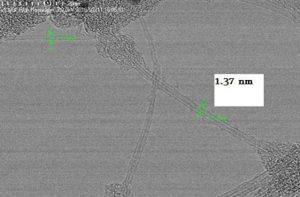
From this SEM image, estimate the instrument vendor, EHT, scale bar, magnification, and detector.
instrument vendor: Hitachi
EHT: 200 kV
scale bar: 20 nm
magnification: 1300 K X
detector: TE